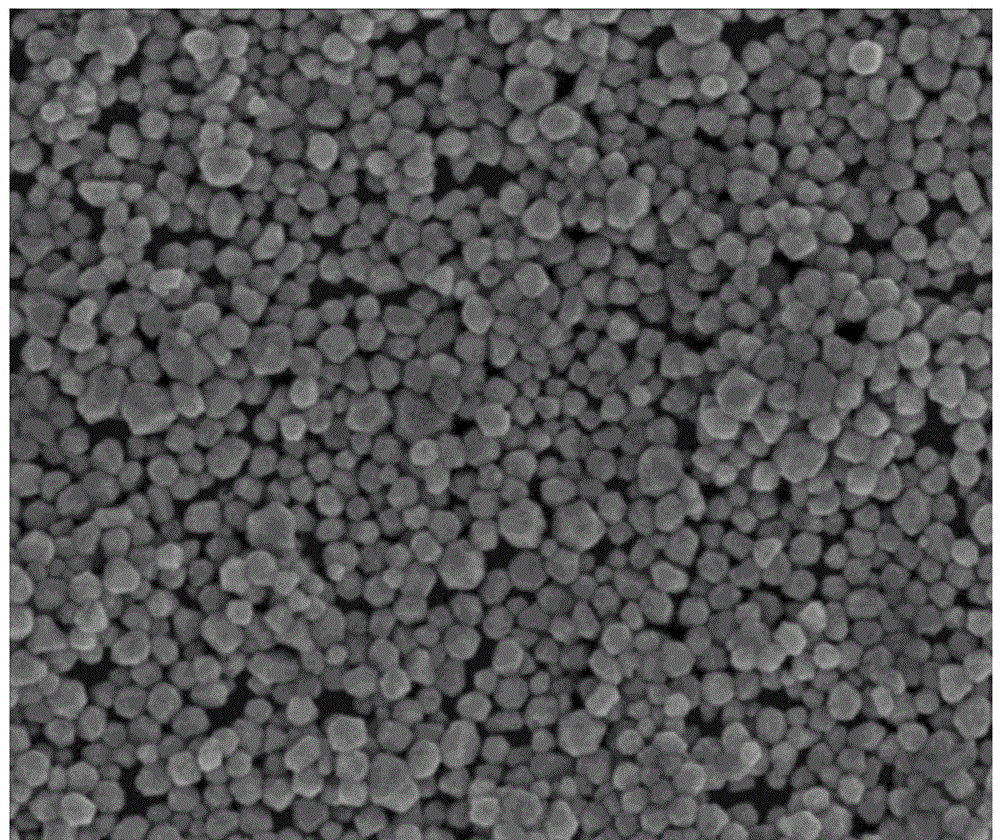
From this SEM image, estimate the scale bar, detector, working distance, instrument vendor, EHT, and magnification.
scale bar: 500 nm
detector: TLD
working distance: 5 mm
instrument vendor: FEI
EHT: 5 kV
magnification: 50 K X